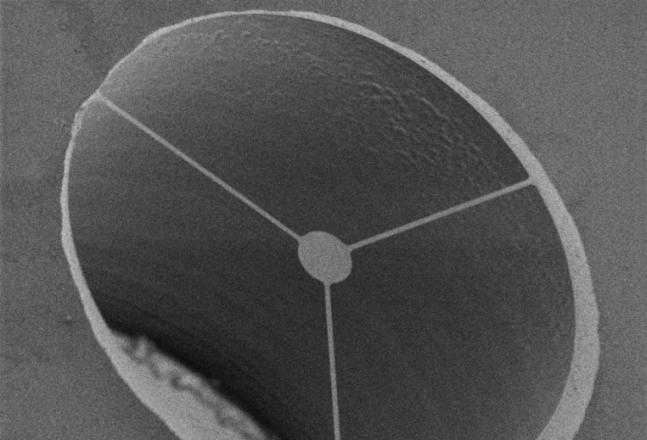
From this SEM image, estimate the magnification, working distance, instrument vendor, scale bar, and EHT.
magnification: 0.3 K X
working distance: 6 mm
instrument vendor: Zeiss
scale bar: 100000 nm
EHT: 7 kV